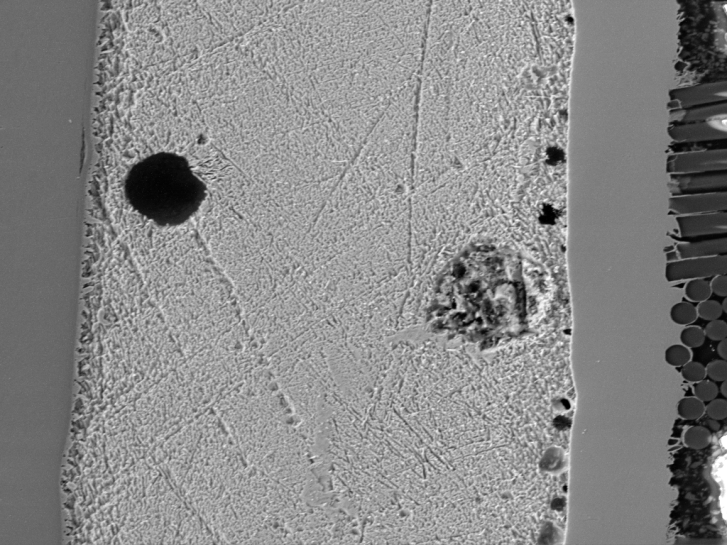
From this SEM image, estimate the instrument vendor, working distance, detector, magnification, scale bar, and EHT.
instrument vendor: Tescan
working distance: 15.81 mm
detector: SE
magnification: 0.976 K X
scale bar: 50000 nm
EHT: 20 kV